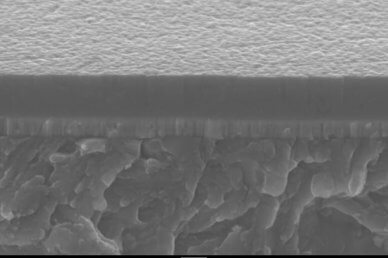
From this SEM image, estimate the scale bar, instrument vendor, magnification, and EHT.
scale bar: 1000 nm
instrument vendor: other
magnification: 8.2 K X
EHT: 20 kV